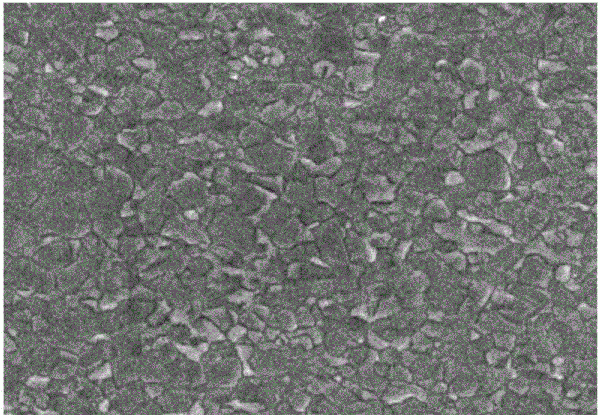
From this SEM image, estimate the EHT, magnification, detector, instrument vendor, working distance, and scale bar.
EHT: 15 kV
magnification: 4 K X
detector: SE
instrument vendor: Hitachi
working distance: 14.3 mm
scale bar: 10000 nm